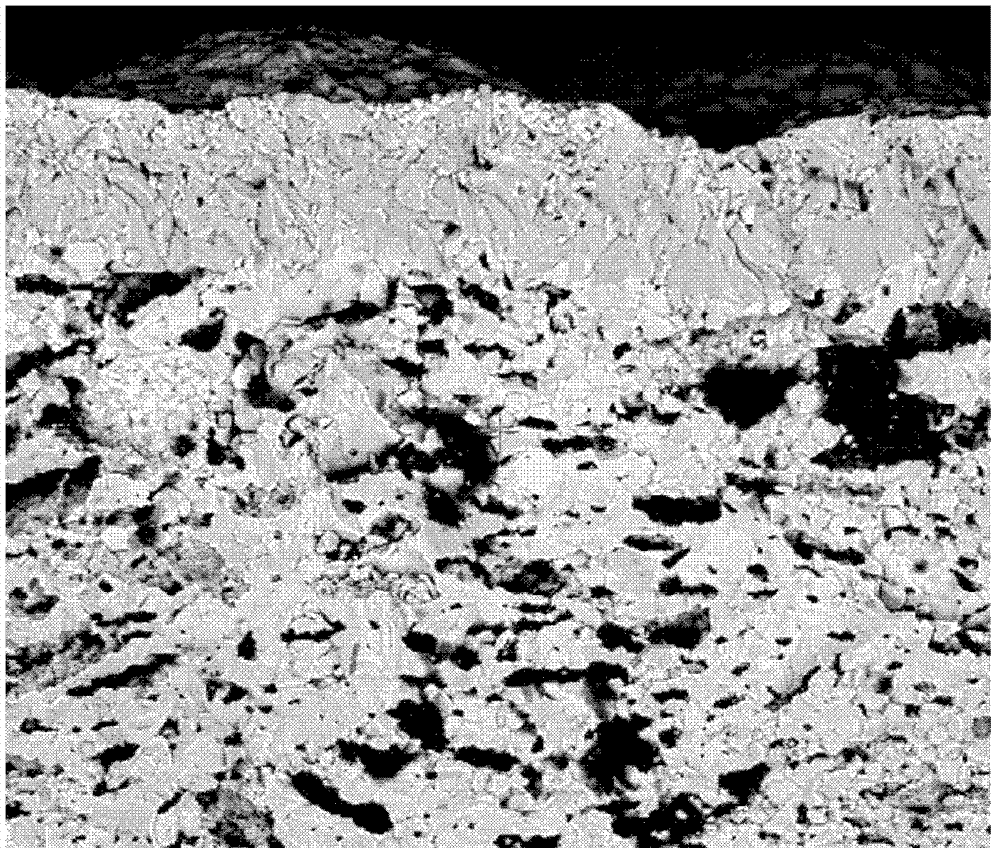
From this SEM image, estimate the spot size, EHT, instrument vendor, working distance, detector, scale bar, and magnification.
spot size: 3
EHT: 10 kV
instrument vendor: FEI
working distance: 7.3 mm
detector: vCD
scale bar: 50000 nm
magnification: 3 K X